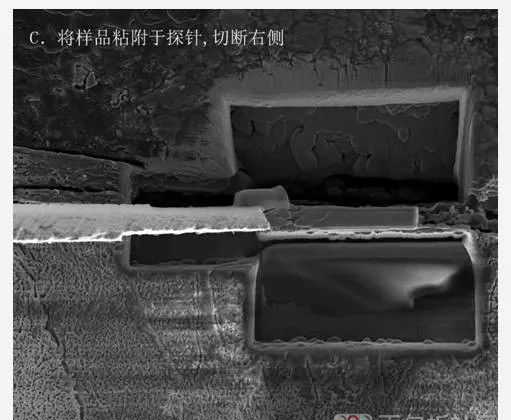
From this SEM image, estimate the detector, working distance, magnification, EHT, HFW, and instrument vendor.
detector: ETD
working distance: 4 mm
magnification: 7 K X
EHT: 5 kV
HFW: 36.6 µm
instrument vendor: FEI